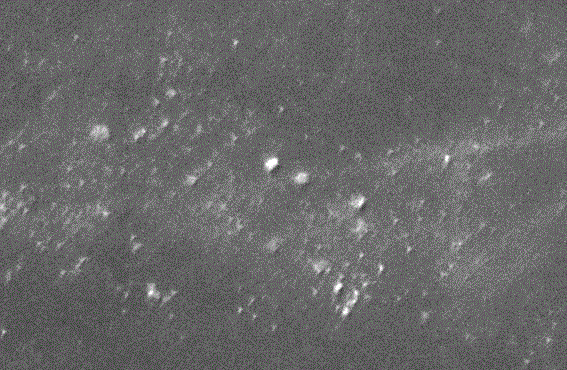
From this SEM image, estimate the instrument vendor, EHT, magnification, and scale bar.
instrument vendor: JEOL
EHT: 20 kV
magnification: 3 K X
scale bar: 5000 nm